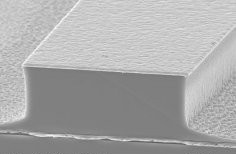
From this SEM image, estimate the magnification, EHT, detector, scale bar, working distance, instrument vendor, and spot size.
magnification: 3.5 K X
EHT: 15 kV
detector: SE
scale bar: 10000 nm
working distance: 16.9 mm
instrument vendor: FEI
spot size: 5